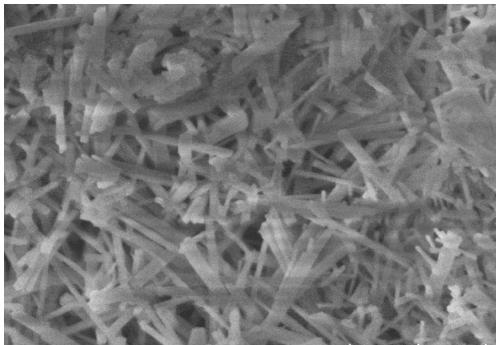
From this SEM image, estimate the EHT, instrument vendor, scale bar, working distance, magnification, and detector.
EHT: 3 kV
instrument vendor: Hitachi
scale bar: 1000 nm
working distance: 9.7 mm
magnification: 35 K X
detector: SE(U)